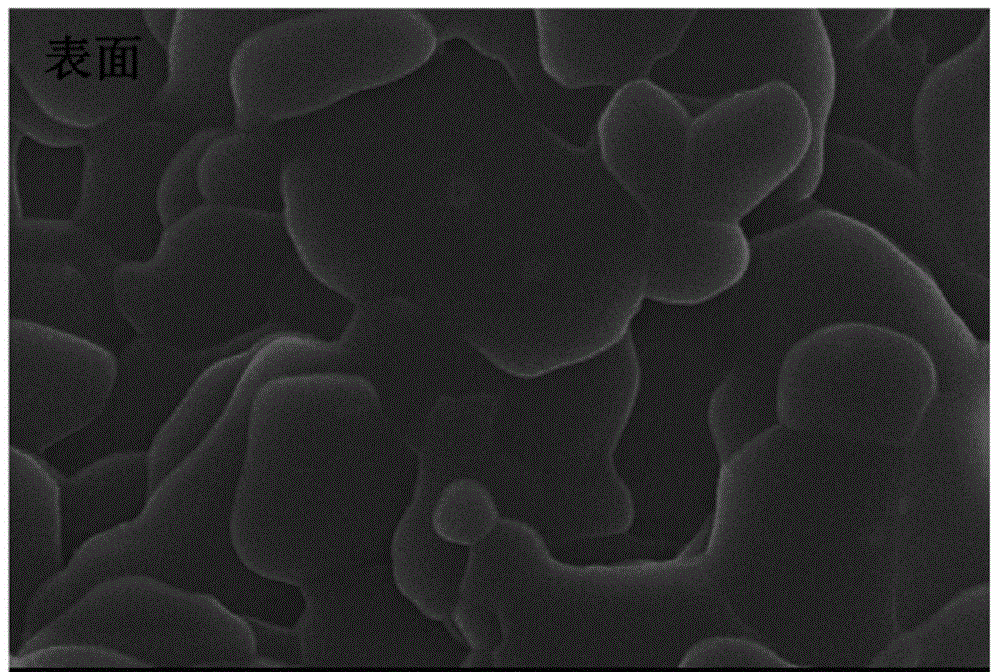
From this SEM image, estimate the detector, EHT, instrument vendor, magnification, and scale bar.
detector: SEI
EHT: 20 kV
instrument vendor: JEOL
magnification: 10 K X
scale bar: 1000 nm